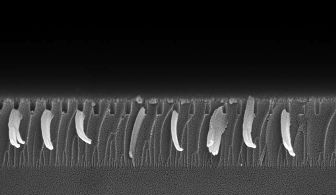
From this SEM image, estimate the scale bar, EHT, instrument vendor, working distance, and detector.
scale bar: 200 nm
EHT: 5 kV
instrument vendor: Zeiss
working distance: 2 mm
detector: InLens